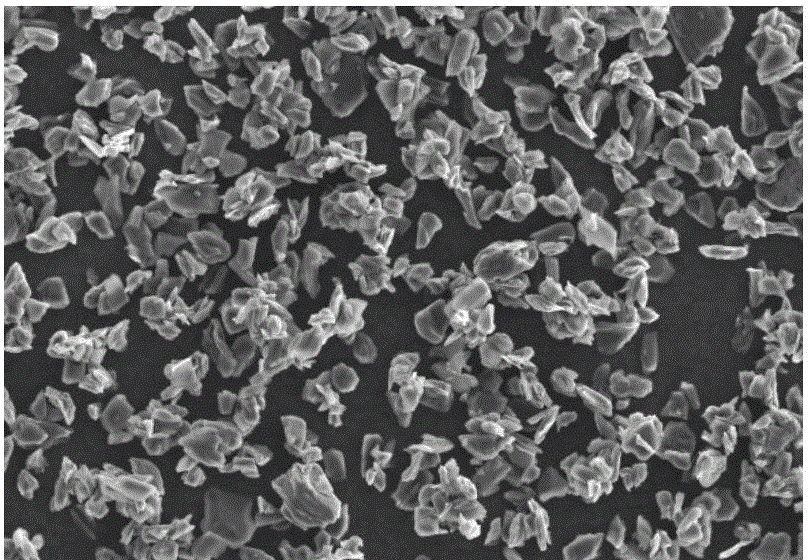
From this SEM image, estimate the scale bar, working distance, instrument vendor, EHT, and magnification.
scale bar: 20000 nm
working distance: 10.2 mm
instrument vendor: other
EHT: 15 kV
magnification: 0.5 K X